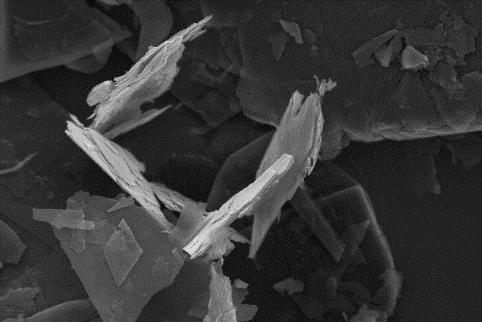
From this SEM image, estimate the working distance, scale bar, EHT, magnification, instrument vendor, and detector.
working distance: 6 mm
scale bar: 10000 nm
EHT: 20 kV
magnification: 2 K X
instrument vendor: Zeiss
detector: SE1